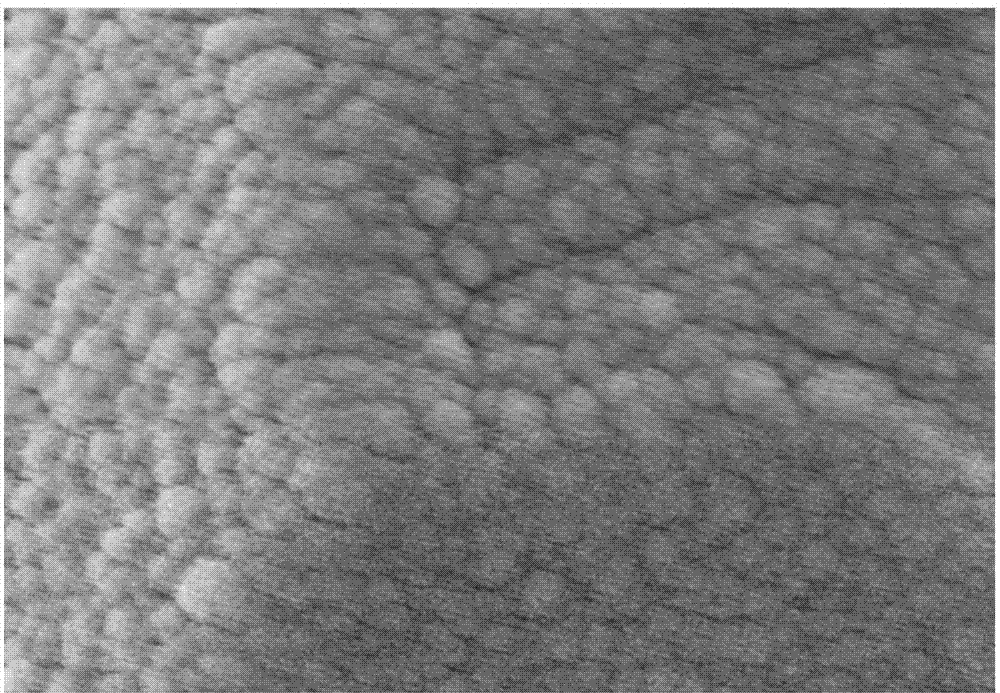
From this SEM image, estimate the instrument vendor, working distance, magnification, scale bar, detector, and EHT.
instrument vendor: Zeiss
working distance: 5.7 mm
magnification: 30 K X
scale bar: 200 nm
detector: InLens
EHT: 5 kV